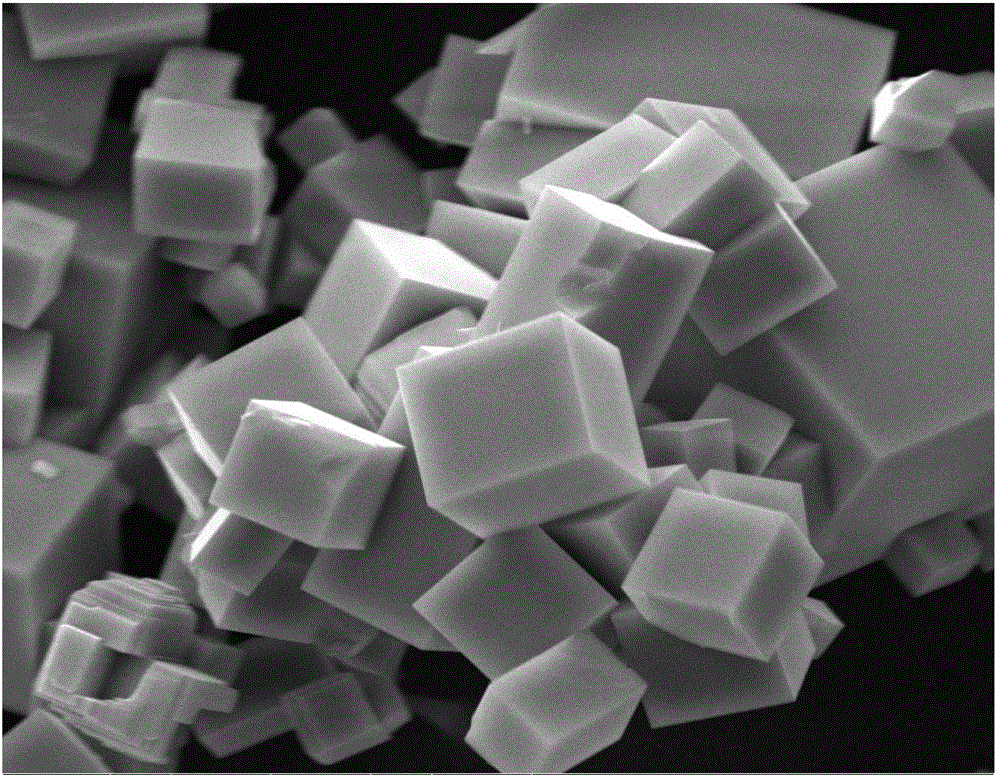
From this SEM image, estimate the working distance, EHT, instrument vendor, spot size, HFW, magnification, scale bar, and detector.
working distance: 7.5 mm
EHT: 25 kV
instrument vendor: FEI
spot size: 4.5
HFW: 59.7 µm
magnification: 5 K X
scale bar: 20000 nm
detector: ETD